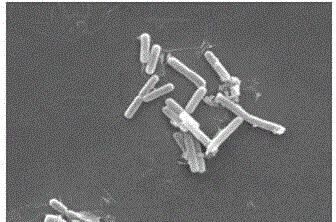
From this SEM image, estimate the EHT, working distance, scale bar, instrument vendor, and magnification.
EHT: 20 kV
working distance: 10.4 mm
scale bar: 5000 nm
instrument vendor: FEI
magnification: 10 K X